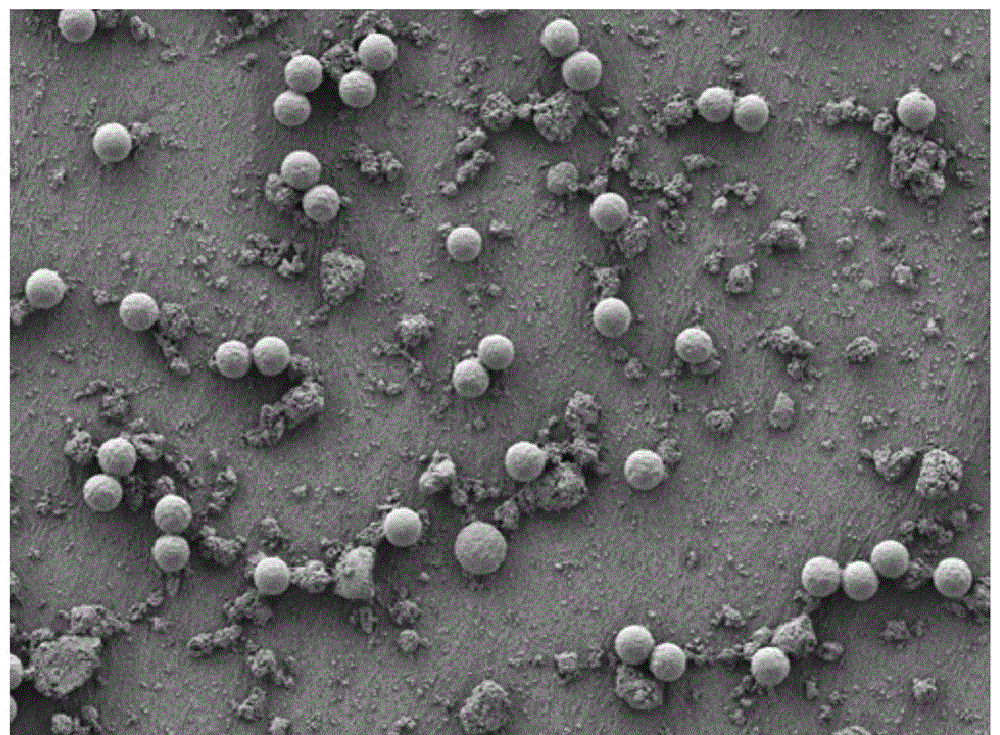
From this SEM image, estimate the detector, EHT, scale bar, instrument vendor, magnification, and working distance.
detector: LED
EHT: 2 kV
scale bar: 10000 nm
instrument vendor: JEOL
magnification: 1 K X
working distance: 4 mm